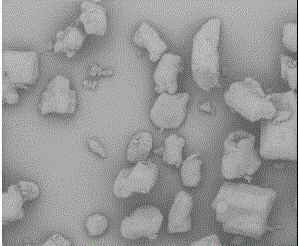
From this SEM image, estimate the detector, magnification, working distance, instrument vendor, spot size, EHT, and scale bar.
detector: Mix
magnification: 0.203 K X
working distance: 11.4 mm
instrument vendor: FEI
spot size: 5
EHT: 10 kV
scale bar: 400000 nm